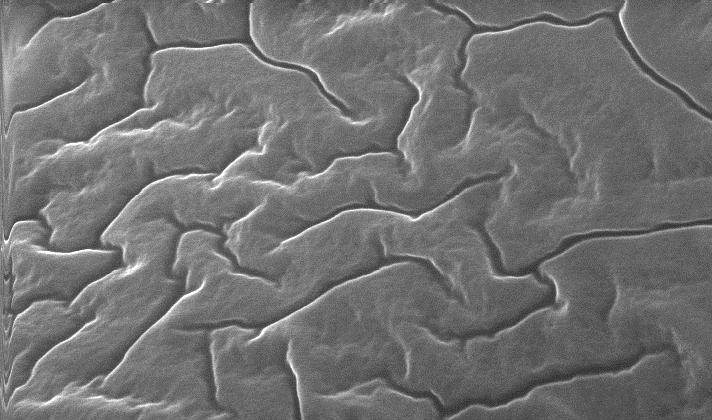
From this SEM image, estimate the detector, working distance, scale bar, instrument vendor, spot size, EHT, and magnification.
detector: SE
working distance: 17.3 mm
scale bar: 2000 nm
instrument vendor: FEI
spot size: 4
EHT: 25 kV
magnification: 10 K X